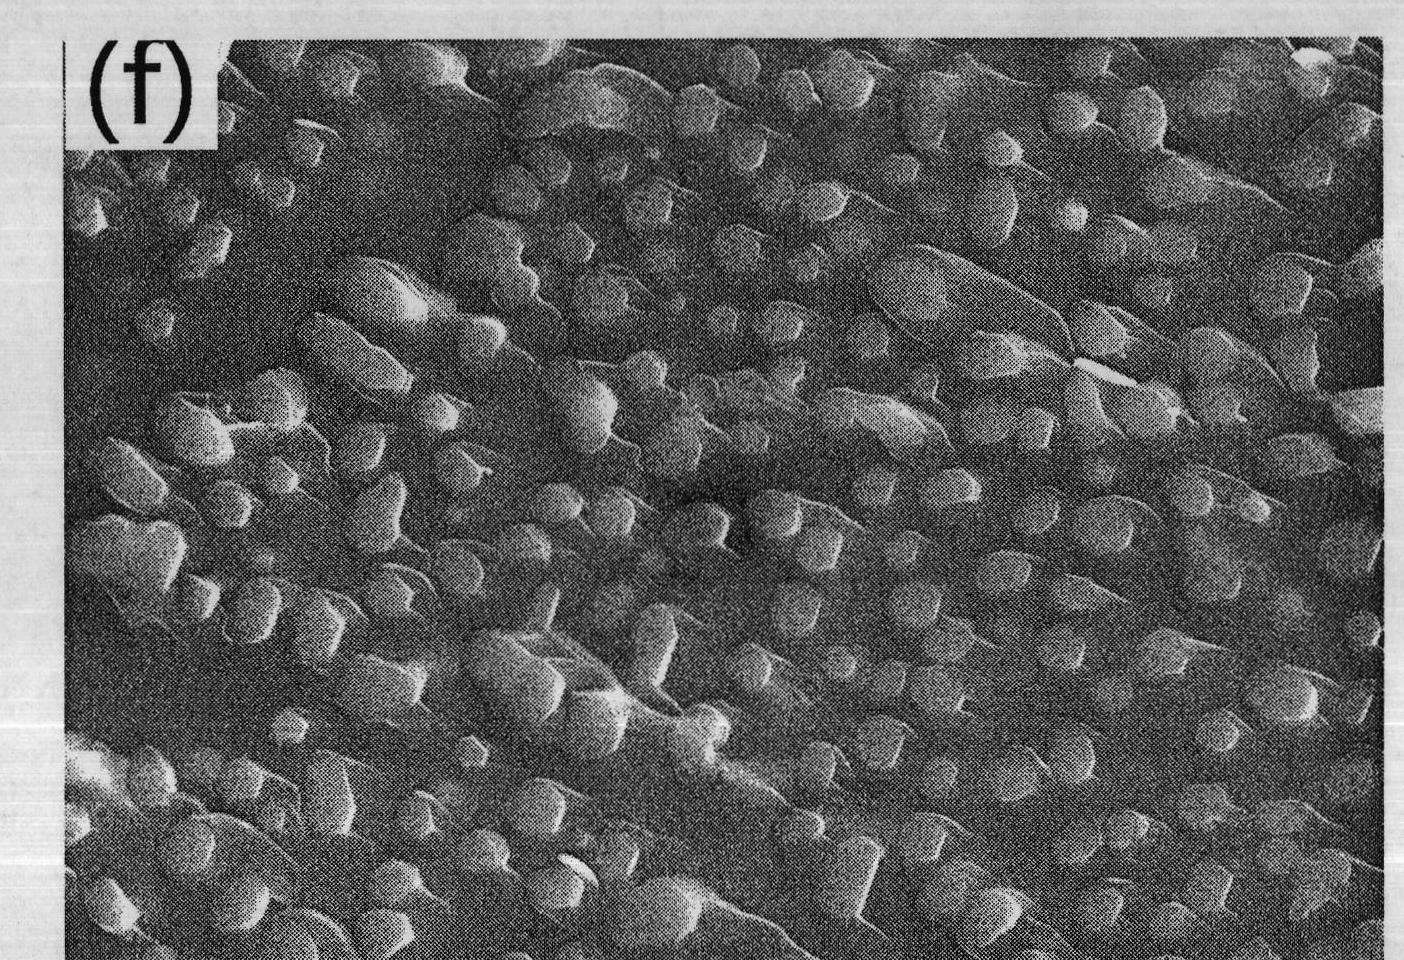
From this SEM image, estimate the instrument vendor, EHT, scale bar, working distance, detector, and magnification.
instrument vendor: JEOL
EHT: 10 kV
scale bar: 1000 nm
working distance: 8.1 mm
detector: SEI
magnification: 10 K X